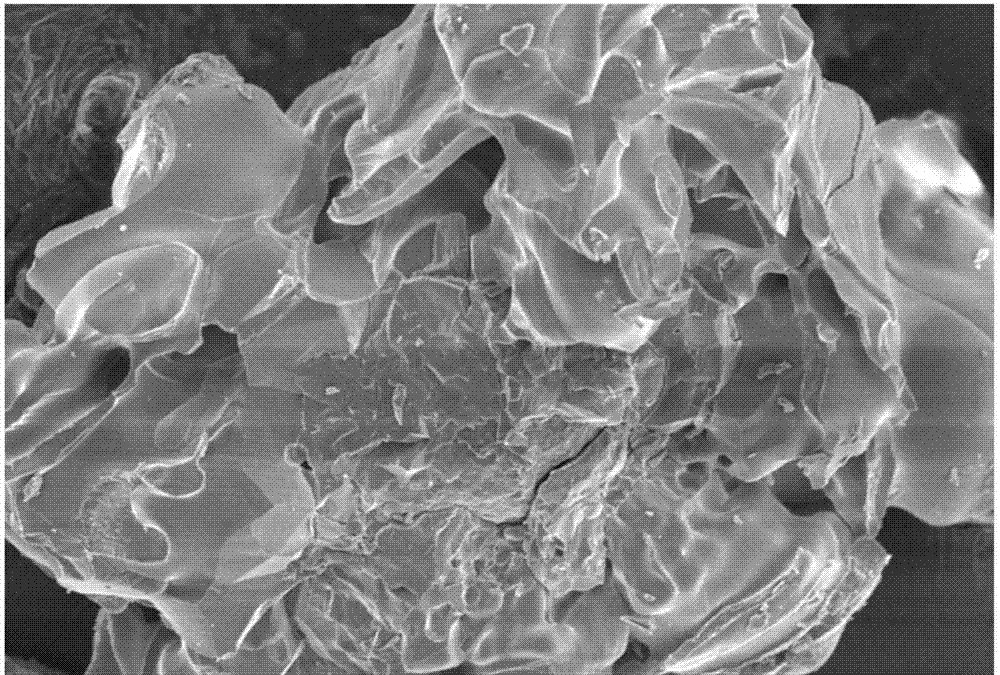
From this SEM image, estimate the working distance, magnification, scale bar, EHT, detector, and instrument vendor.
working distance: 16 mm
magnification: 1.2 K X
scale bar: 10000 nm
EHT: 5 kV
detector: SEI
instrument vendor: JEOL